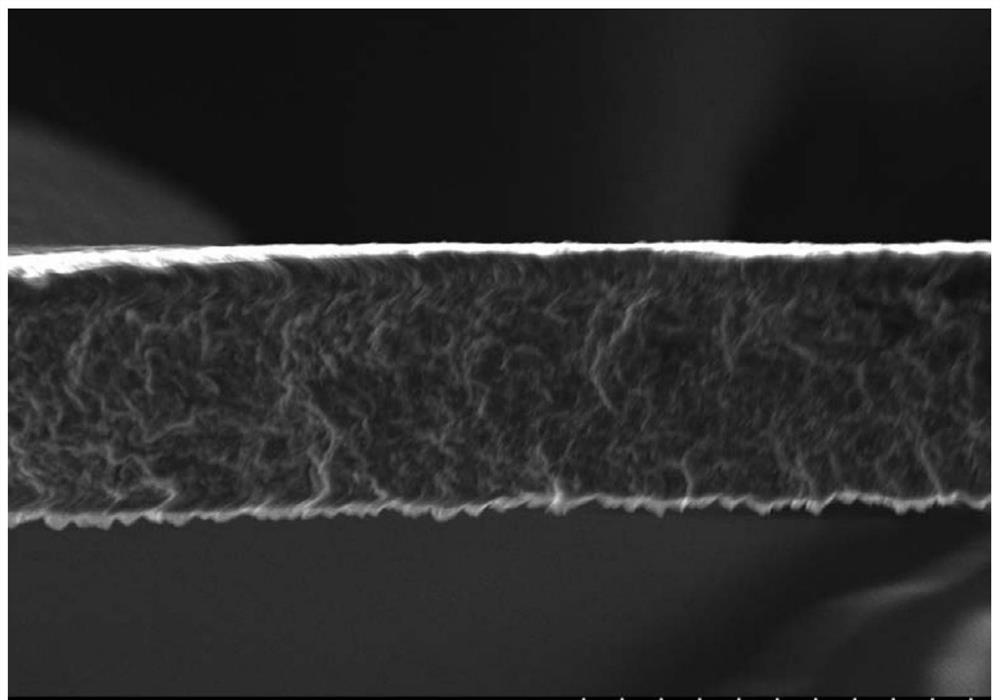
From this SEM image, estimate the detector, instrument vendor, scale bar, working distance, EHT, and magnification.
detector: SE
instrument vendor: Hitachi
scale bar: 50000 nm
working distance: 10 mm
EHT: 15 kV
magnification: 1 K X